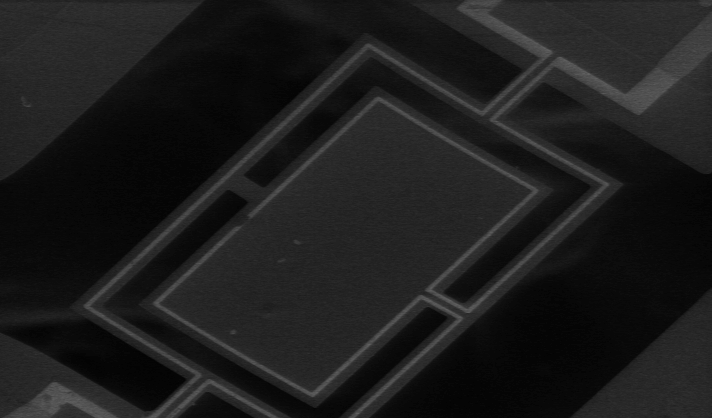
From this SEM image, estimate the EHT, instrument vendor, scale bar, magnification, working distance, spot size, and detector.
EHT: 10 kV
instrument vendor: FEI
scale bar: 50000 nm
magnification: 0.446 K X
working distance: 15 mm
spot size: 3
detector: SE